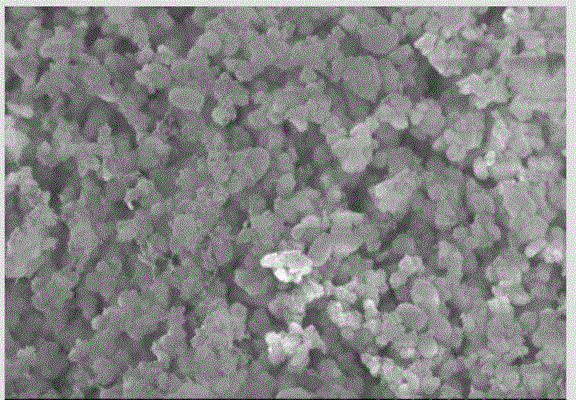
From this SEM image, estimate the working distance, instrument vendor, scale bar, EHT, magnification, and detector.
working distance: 9.6 mm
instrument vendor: Hitachi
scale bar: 20000 nm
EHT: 15 kV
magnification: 2 K X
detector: SE